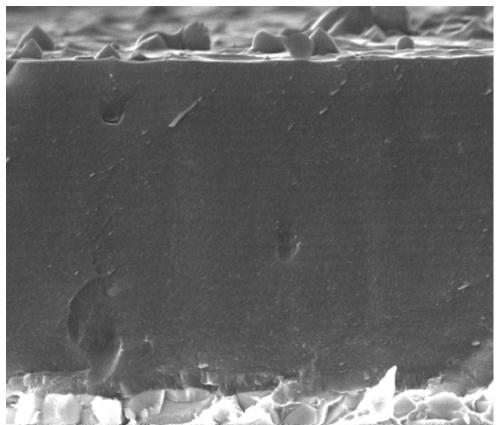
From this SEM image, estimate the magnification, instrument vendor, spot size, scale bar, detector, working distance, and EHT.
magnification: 50 K X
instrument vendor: FEI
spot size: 3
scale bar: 2000 nm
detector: TLD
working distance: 5.8 mm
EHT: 10 kV